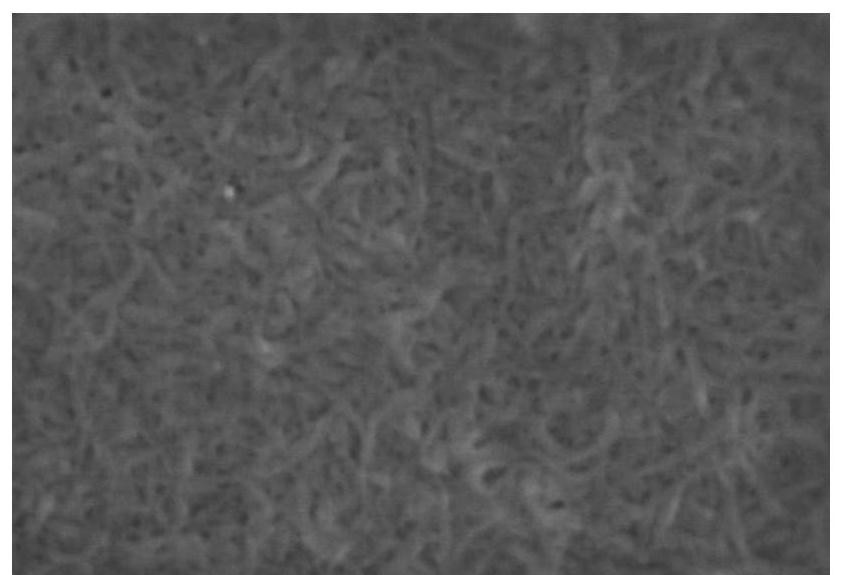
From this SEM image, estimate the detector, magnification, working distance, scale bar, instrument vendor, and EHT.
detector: SE(UL)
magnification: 100 K X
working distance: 6 mm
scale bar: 500 nm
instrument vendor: Hitachi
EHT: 5 kV